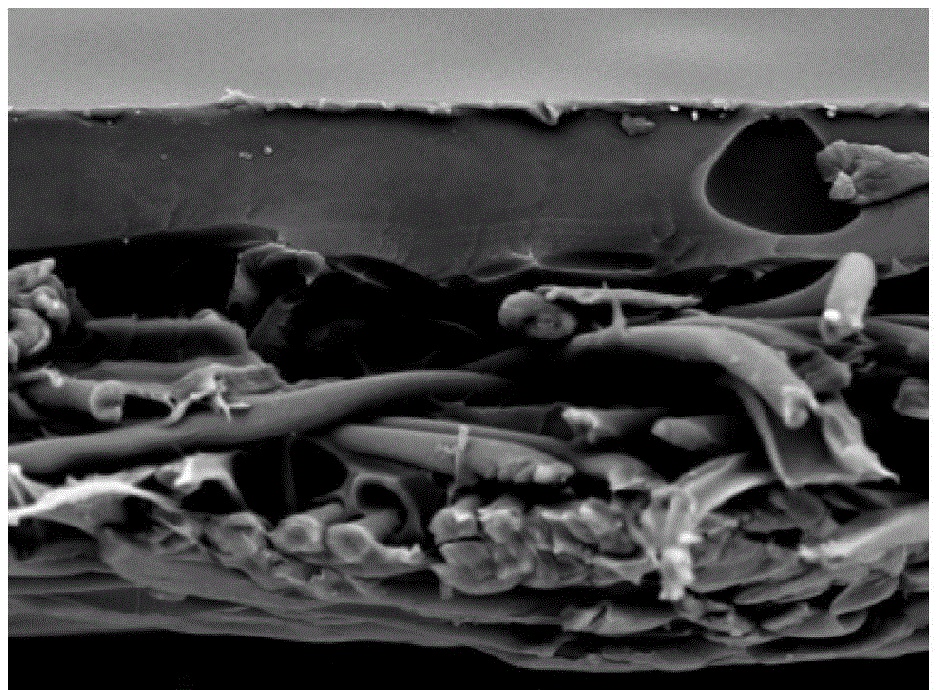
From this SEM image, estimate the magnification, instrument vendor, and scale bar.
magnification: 1 K X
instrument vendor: Hitachi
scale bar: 100000 nm